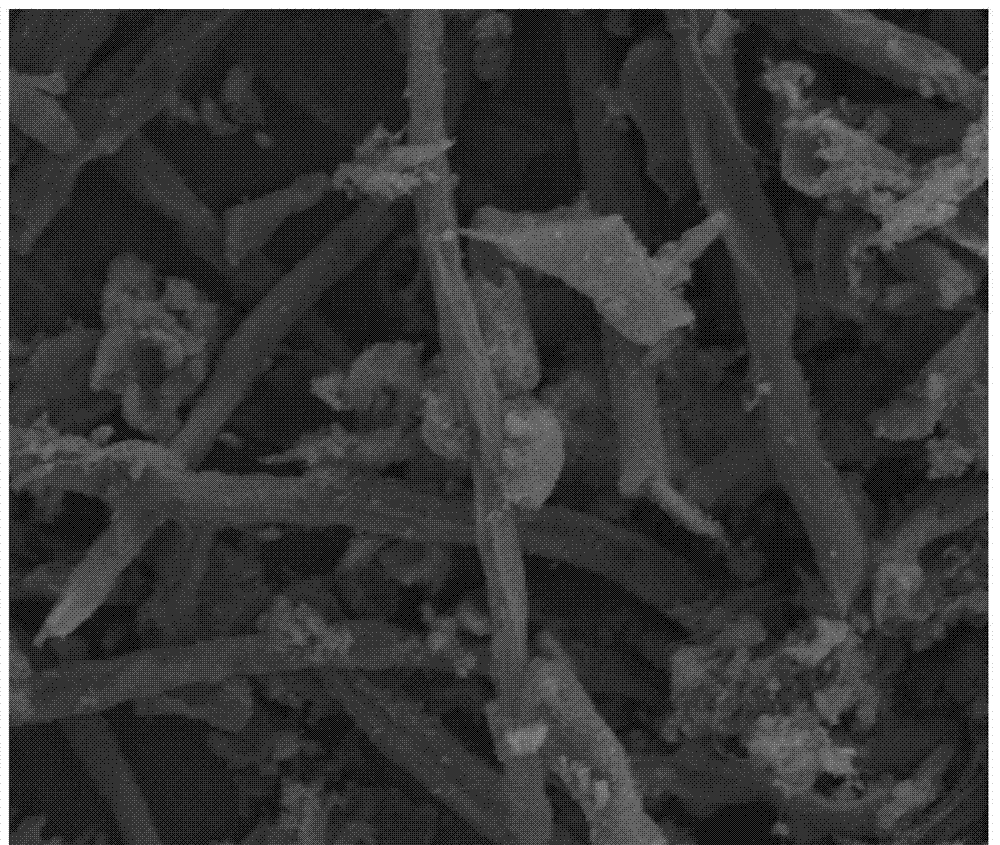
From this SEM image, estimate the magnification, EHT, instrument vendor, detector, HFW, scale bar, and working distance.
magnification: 5 K X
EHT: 10 kV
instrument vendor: FEI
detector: ETD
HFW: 48 µm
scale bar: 10000 nm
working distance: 5.7 mm